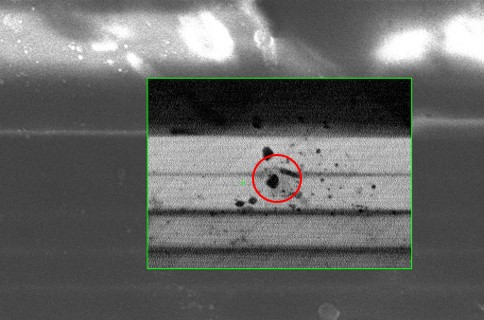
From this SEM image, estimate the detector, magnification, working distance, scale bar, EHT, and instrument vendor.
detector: InLens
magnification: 8.75 K X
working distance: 4.7 mm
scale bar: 2000 nm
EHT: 3 kV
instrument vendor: Zeiss